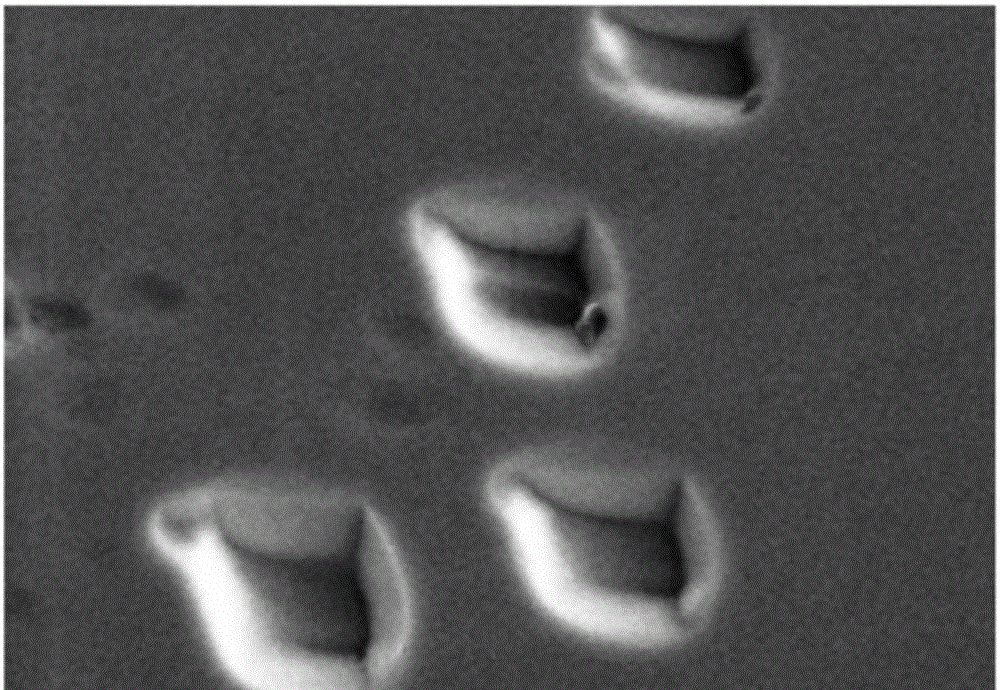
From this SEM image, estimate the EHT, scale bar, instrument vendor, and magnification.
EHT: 10 kV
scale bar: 5000 nm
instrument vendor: Hitachi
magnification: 8 K X